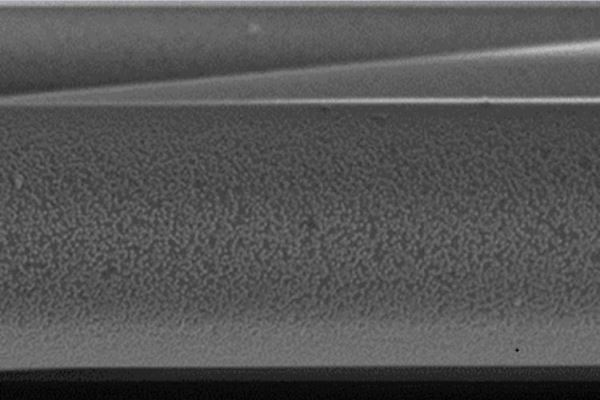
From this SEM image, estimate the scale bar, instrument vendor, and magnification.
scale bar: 1000 nm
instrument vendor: JEOL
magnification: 10 K X